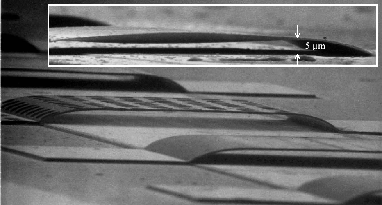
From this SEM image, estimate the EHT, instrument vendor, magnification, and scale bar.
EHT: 30 kV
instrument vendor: JEOL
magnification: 0.9 K X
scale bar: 33300 nm